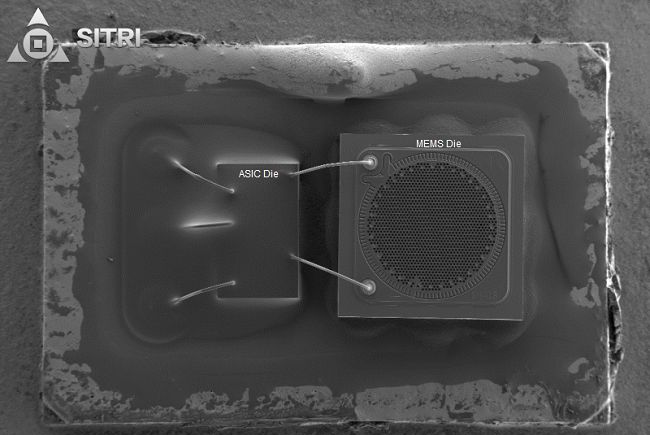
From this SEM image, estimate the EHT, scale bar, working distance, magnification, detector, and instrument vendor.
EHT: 5 kV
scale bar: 100000 nm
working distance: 7.2 mm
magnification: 0.1 K X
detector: SE2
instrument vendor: Zeiss